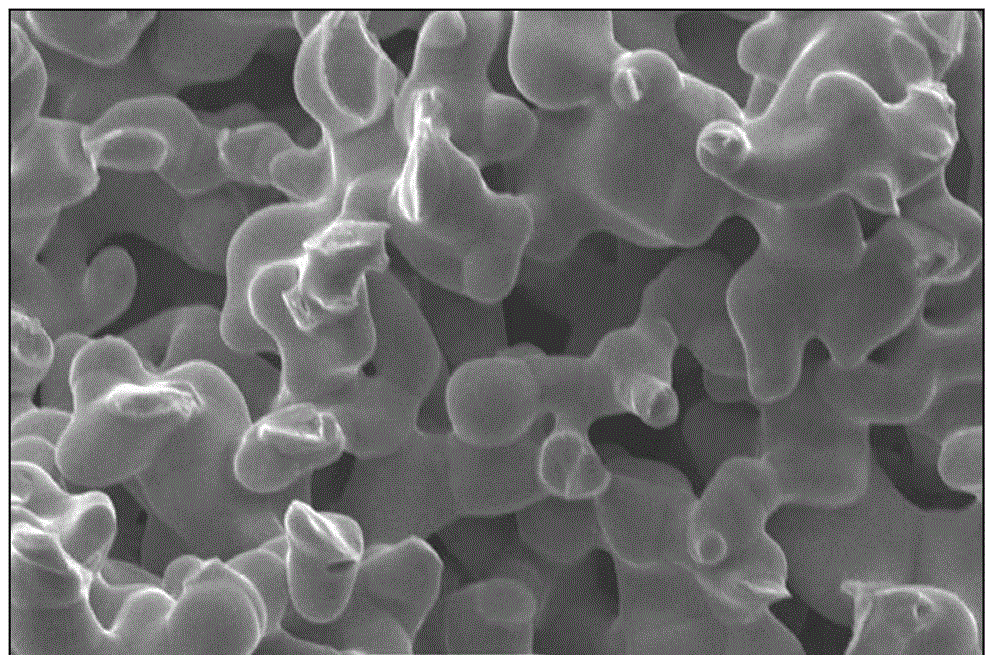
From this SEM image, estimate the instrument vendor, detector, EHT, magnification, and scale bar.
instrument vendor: JEOL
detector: SEI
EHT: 20 kV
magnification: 2.5 K X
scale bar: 10000 nm